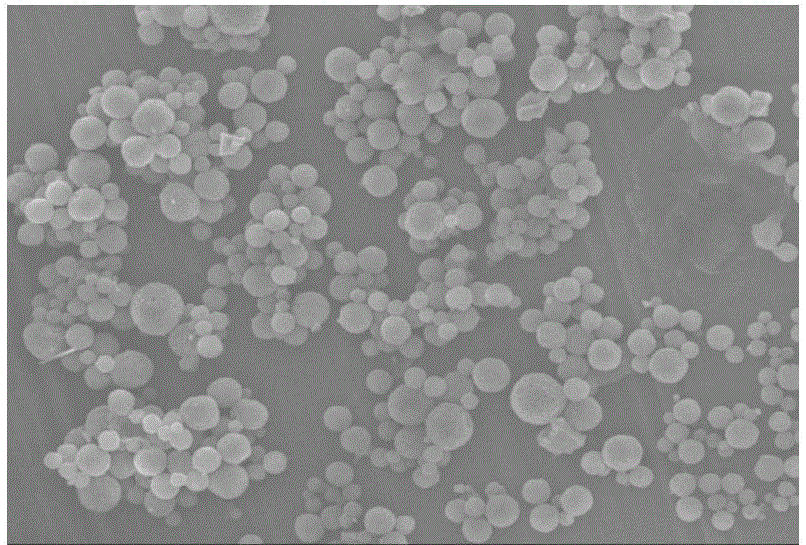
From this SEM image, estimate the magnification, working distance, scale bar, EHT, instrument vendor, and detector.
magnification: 1 K X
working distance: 17 mm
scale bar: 10000 nm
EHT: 15 kV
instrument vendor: JEOL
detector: SEI